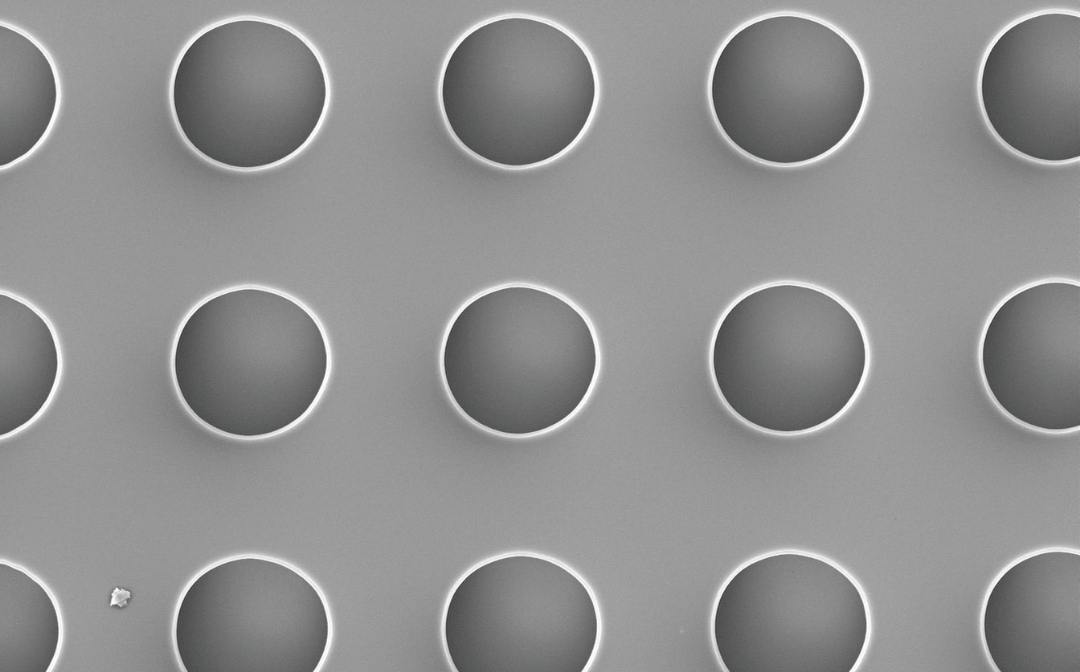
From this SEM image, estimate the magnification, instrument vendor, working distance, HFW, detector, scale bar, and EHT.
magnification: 2 K X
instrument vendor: Thermo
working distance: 9.437 mm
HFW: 63.5 µm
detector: SED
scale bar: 20000 nm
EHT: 5 kV